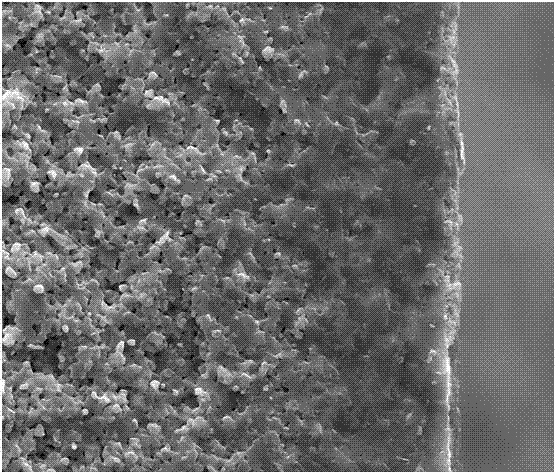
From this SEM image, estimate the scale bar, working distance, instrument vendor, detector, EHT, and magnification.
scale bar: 20000 nm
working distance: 11.9 mm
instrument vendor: FEI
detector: SE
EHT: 30 kV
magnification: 5 K X